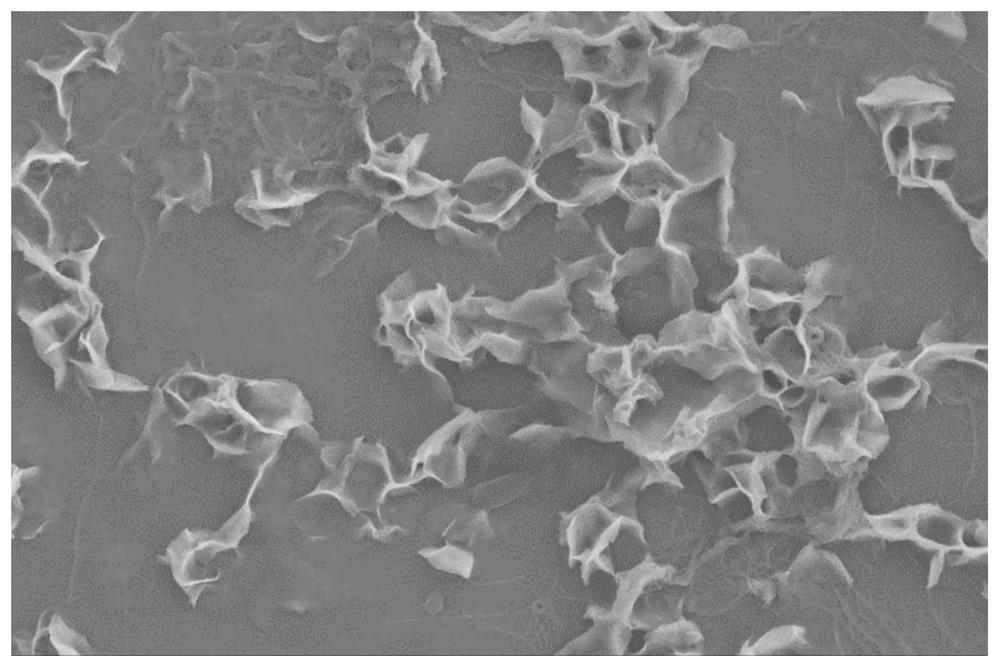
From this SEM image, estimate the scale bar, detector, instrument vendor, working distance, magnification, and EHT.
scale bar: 2000 nm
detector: SE2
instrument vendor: Zeiss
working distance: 11.2 mm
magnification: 5 K X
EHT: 5 kV